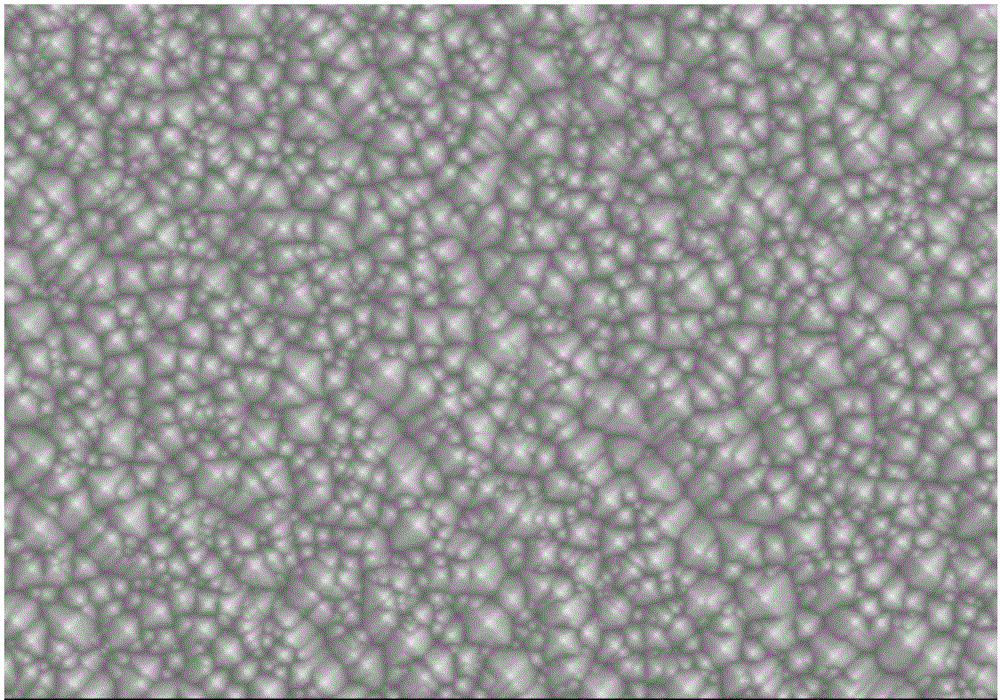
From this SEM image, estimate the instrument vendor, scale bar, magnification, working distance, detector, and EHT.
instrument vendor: Hitachi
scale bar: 50000 nm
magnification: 1 K X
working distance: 13.8 mm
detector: SE(M)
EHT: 15 kV